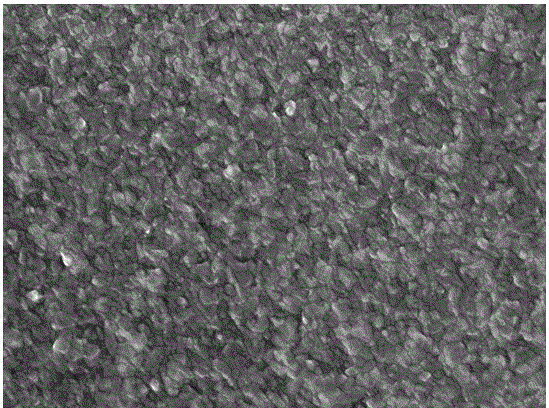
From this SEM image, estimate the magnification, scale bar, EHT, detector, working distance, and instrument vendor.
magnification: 50 K X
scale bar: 100 nm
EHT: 15 kV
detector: SEI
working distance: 7 mm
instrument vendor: JEOL